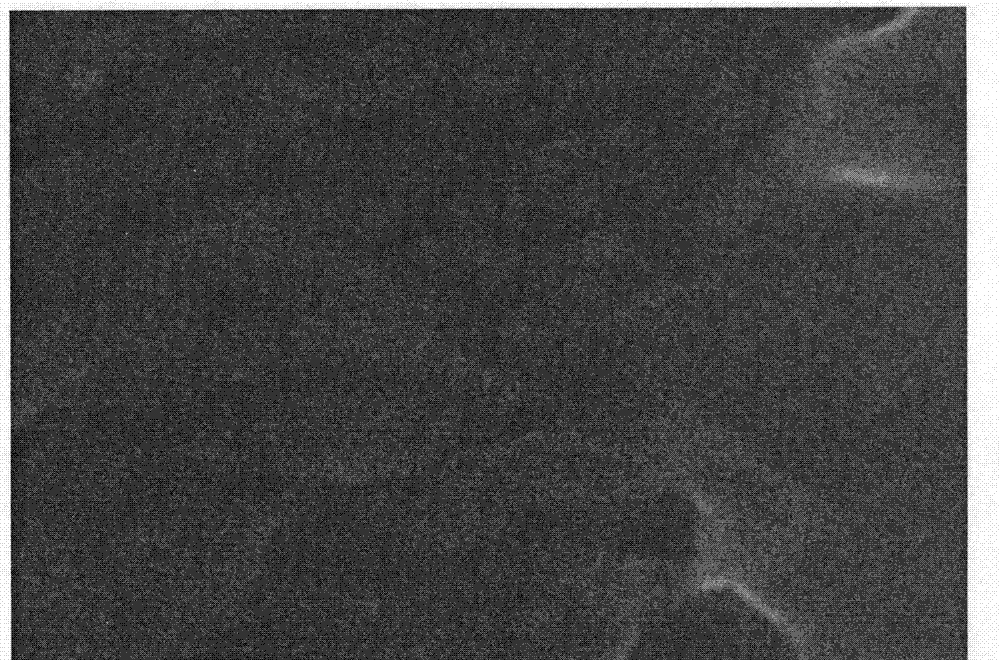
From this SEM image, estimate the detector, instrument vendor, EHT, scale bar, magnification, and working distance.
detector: SE(M)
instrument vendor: Hitachi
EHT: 10 kV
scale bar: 200 nm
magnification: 200 K X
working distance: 5.8 mm